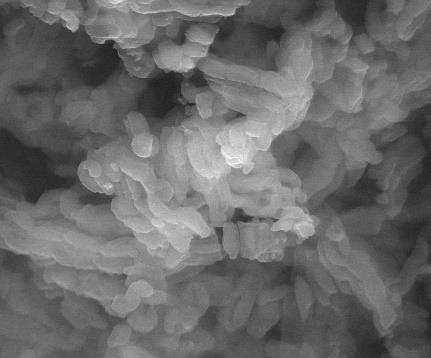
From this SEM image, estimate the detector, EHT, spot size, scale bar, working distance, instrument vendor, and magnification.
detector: LFD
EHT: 30 kV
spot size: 4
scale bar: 5000 nm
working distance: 10 mm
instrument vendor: FEI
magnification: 20 K X